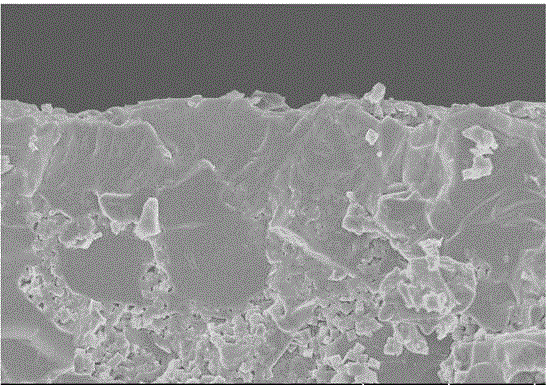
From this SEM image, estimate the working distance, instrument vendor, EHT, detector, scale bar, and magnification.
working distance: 9.5 mm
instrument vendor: Hitachi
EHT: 5 kV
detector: SE(UL)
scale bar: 10000 nm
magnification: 4 K X